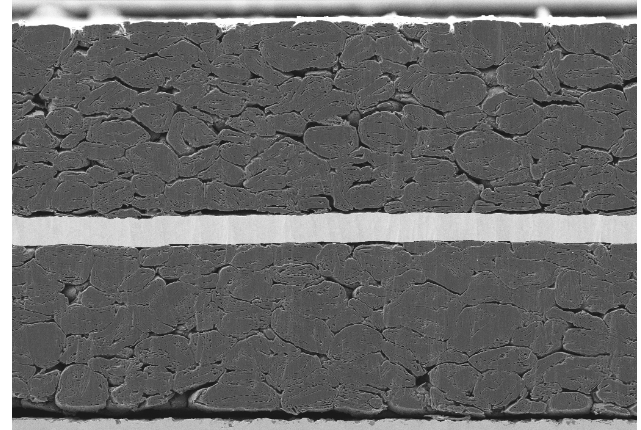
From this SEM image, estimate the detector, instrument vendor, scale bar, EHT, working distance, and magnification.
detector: SE2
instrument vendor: Zeiss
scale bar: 10000 nm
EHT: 5 kV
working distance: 5.1 mm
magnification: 0.55 K X